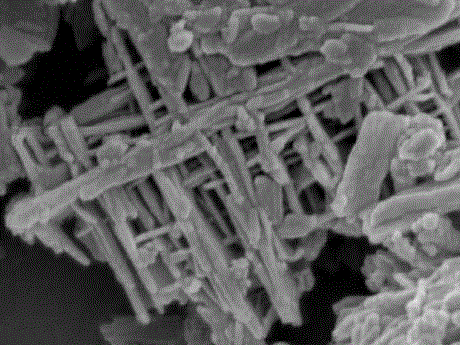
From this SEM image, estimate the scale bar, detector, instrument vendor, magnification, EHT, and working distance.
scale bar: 100 nm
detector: SEI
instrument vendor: JEOL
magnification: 60 K X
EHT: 5 kV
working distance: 8 mm